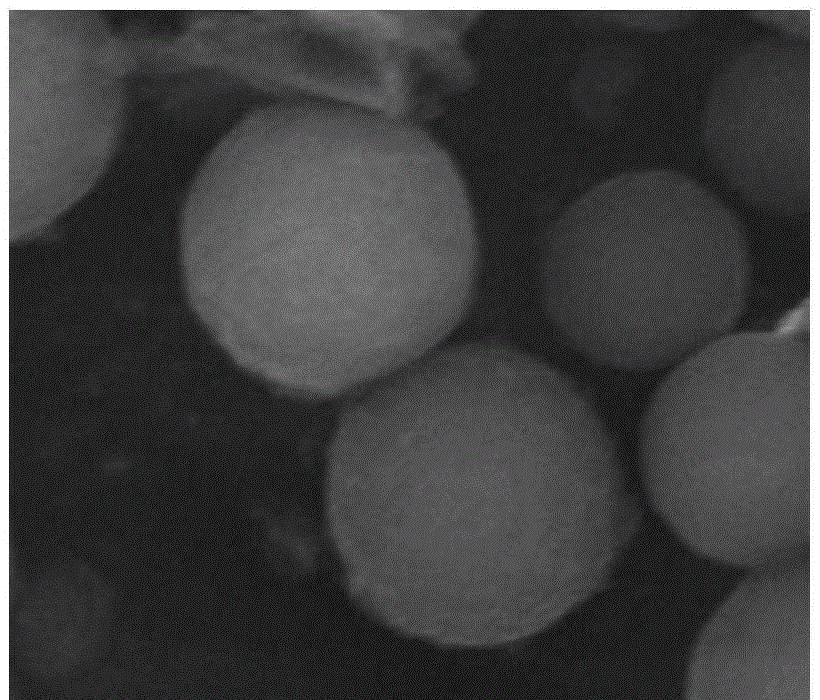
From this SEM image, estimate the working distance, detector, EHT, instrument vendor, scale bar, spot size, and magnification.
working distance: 21.3 mm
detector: ETD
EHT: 30 kV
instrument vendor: FEI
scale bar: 3000 nm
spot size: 3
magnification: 30 K X